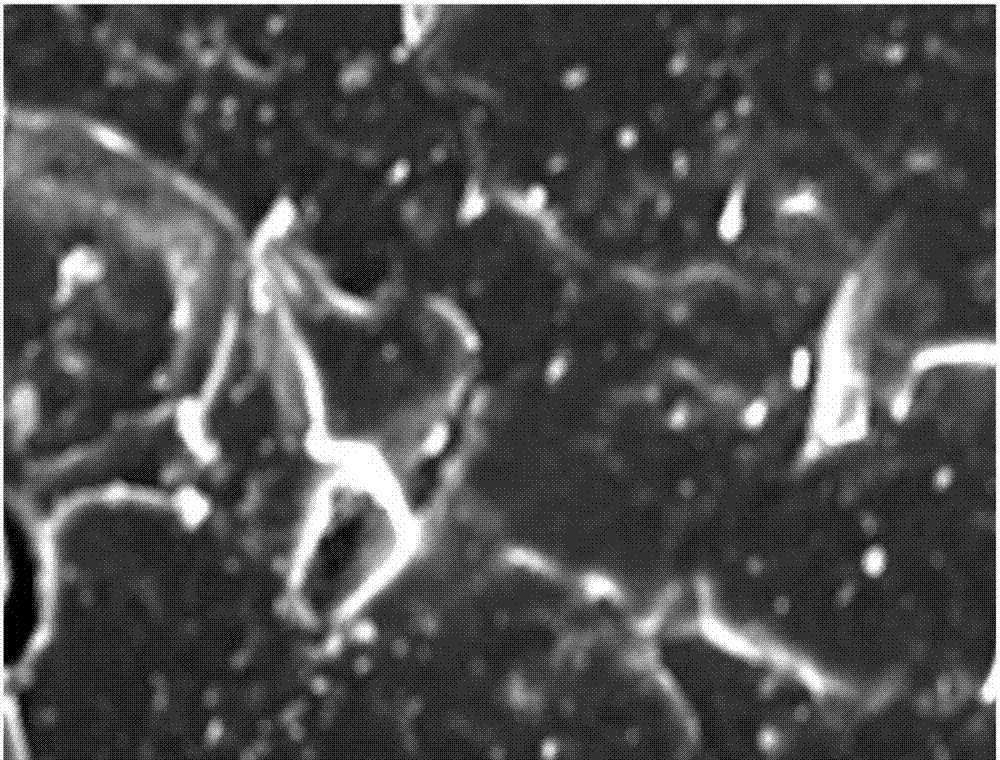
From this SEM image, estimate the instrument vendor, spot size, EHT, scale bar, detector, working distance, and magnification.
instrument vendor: FEI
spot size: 3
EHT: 15 kV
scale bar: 50000 nm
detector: ETD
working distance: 5 mm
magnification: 2 K X